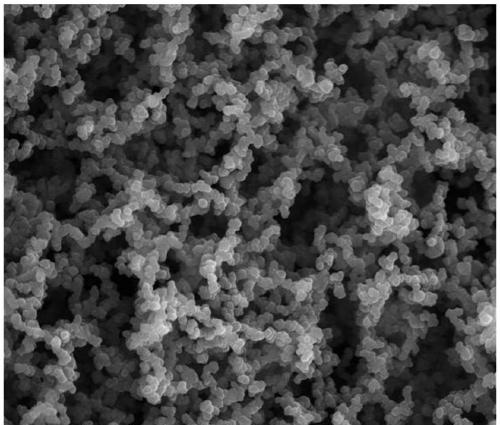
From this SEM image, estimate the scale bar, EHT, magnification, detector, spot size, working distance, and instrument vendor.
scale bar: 4000 nm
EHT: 15 kV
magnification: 20 K X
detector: SE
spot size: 3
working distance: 6.4 mm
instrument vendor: FEI